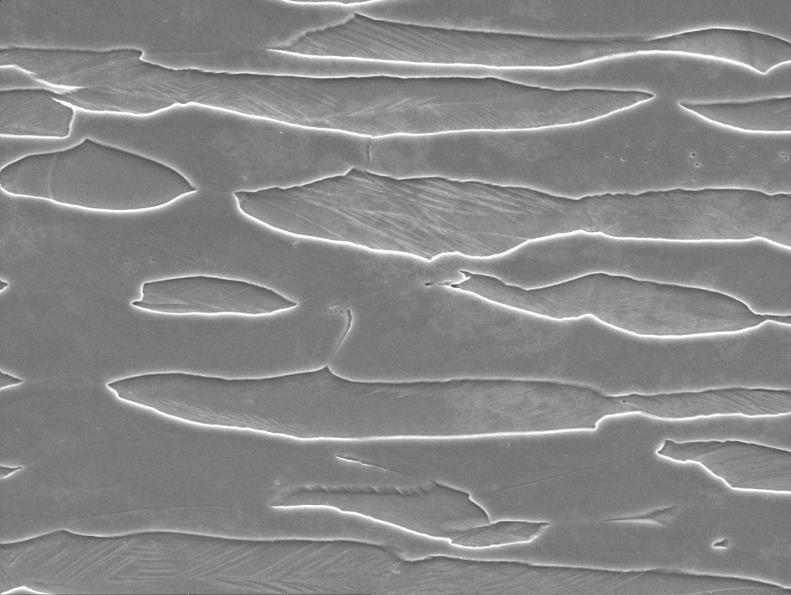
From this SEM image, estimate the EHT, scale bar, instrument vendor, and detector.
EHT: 20 kV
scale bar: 10000 nm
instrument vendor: JEOL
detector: SEI